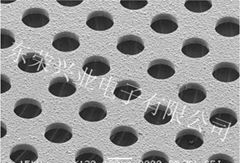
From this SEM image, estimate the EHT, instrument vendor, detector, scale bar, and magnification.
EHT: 15 kV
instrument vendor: JEOL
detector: SEI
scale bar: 100000 nm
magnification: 0.13 K X